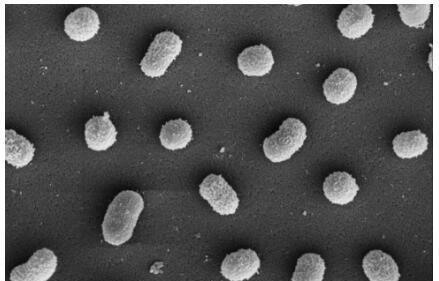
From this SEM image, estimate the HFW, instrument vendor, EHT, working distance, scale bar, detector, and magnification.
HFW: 8.29 µm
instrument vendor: FEI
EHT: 5 kV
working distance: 4.1 mm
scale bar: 2000 nm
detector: ETD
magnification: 24.991 K X